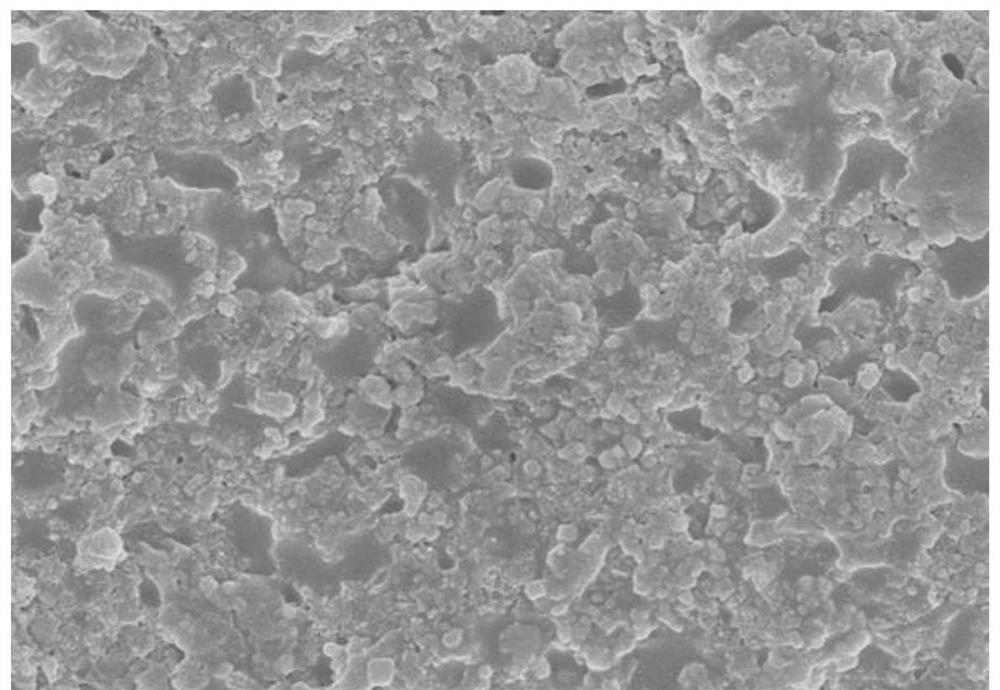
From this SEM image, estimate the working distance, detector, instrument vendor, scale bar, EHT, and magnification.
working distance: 8.4 mm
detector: SE(M)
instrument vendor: Hitachi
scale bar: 5000 nm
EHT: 5 kV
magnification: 10 K X